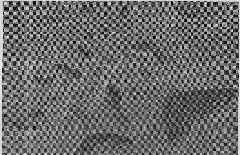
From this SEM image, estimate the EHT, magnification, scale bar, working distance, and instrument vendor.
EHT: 10 kV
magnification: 0.5 K X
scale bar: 100000 nm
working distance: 12.4 mm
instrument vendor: Hitachi